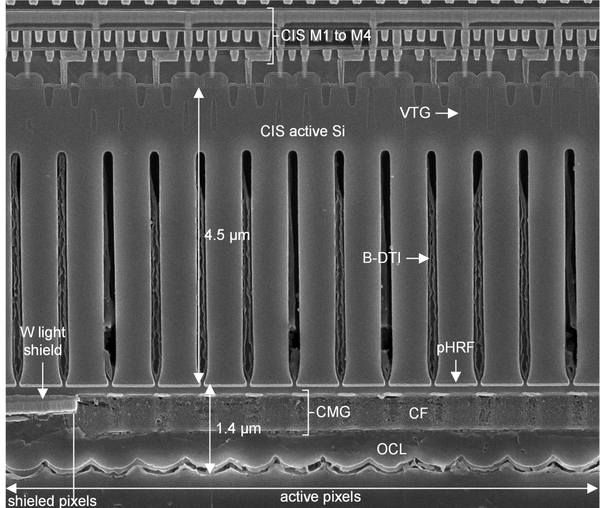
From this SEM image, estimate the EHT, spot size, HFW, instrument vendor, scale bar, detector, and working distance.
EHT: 5 kV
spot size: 2.5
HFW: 9.07 µm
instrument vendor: FEI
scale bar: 3000 nm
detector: TLD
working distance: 5.5 mm